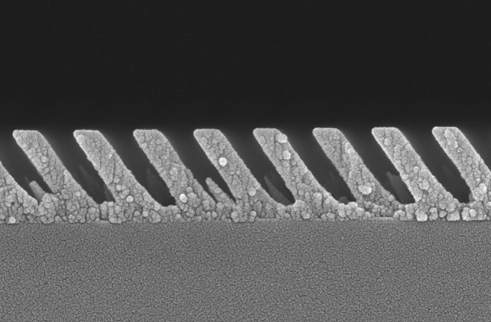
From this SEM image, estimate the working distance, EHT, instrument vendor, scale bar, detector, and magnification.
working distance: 3 mm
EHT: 5 kV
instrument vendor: Zeiss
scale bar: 200 nm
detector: InLens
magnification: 100 K X